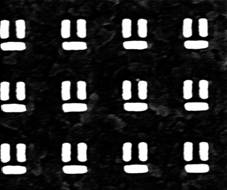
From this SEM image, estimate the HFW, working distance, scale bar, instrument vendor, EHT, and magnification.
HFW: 2.98 µm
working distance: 5.6 mm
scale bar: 1000 nm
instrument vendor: FEI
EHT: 5 kV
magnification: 50 K X